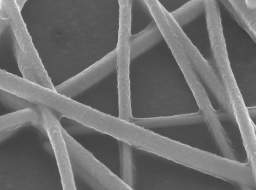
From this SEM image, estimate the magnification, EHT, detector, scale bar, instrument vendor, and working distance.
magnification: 60 K X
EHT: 8 kV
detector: SEI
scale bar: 100 nm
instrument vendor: JEOL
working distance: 6.5 mm